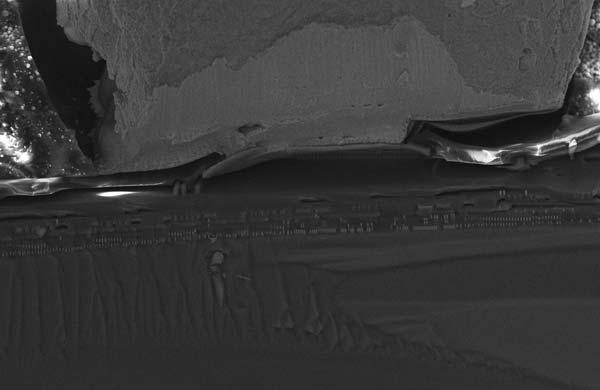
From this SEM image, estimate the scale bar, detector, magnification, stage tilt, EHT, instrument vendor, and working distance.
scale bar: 10000 nm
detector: InLens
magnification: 2 K X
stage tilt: -15°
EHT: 15 kV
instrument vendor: Zeiss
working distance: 4 mm